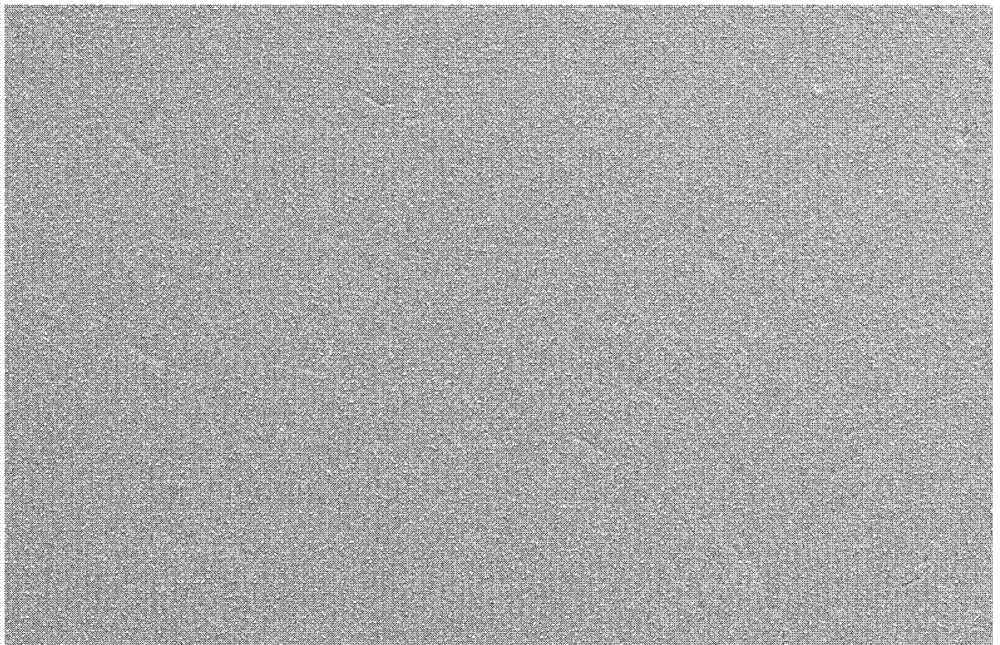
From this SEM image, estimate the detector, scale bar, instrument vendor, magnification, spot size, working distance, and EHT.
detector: SE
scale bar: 100000 nm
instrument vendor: FEI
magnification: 0.5 K X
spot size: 3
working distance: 6.2 mm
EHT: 10 kV